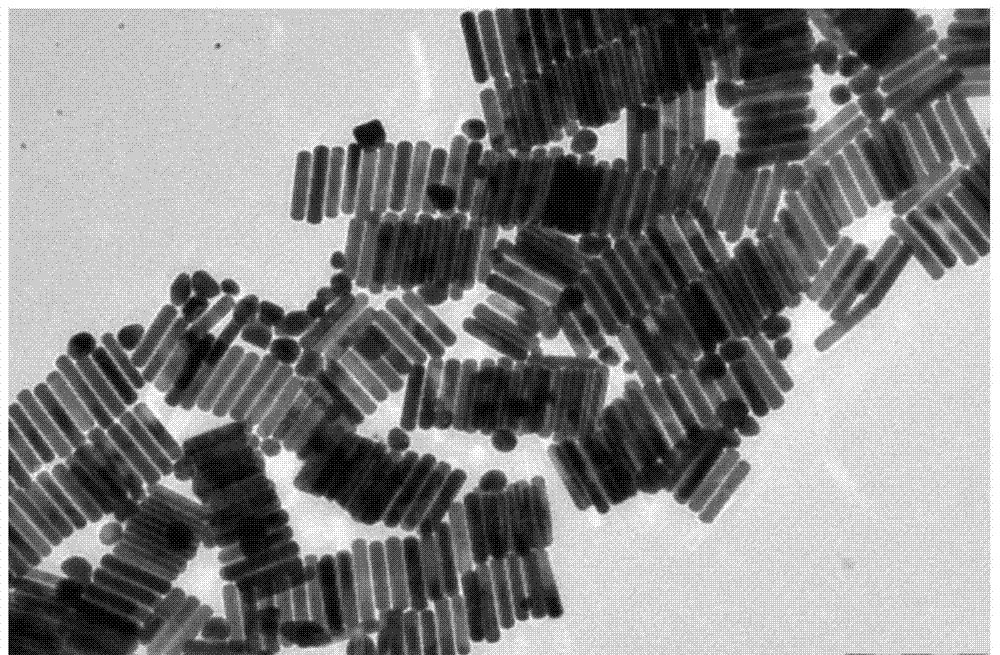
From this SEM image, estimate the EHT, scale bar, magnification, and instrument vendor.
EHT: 100 kV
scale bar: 200 nm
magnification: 40 K X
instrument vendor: JEOL